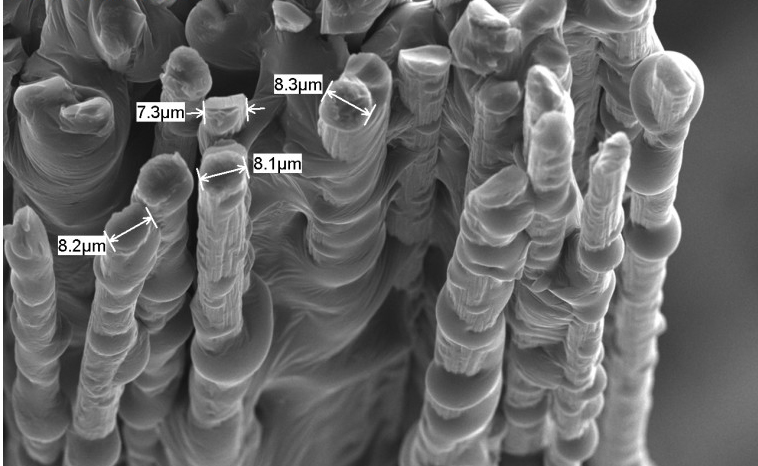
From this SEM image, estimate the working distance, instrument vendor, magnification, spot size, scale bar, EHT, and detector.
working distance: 10 mm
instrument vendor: JEOL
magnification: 1 K X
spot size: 48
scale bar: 10000 nm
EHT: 15 kV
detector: SEI